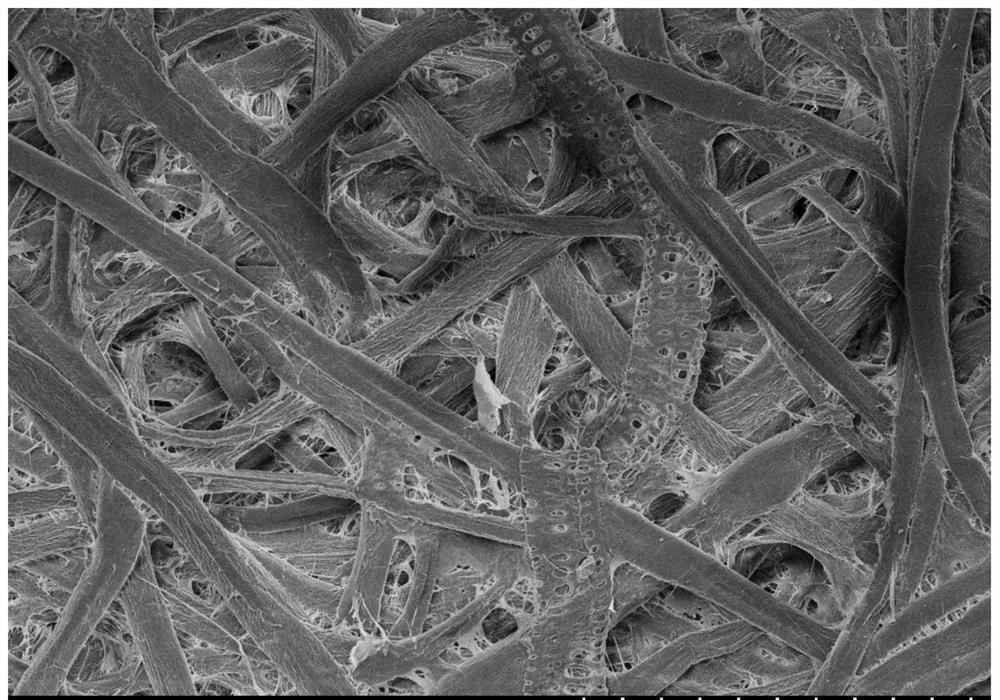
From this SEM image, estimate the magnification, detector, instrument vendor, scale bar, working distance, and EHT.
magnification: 0.5 K X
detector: SE(M)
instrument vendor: Hitachi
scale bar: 100000 nm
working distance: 12.1 mm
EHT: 5 kV